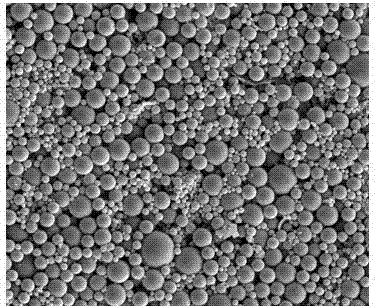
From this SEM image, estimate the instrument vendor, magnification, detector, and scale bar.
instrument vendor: FEI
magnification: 10 K X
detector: ETD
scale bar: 10000 nm